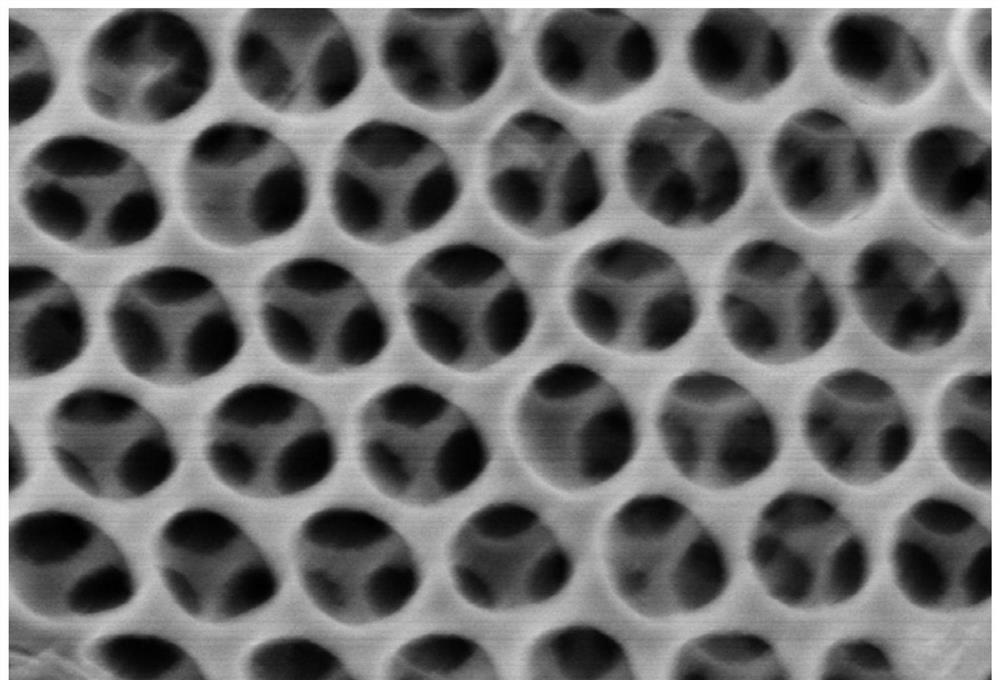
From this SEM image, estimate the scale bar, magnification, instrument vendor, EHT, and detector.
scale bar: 1000 nm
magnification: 40 K X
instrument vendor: Hitachi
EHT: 3 kV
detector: SE(U)